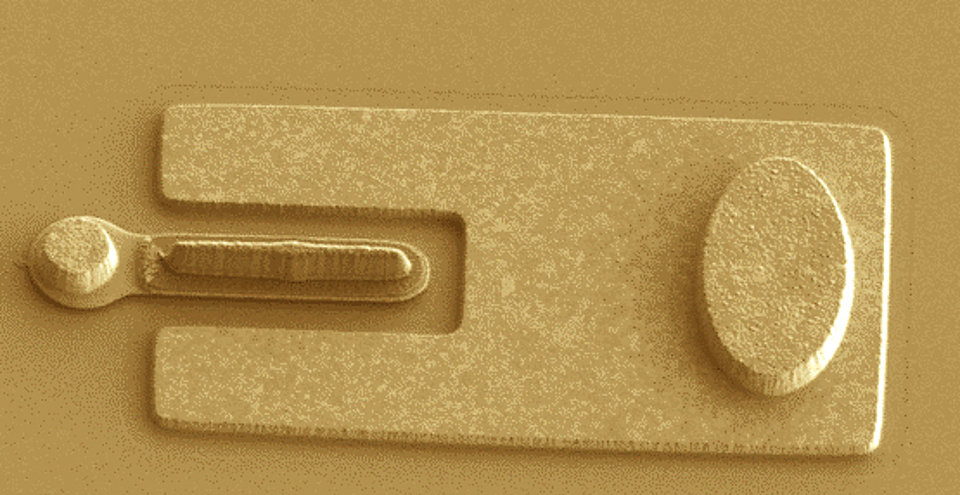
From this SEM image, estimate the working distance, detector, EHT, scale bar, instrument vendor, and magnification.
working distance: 7.9 mm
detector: SE(L)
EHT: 1 kV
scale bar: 5000 nm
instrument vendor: Hitachi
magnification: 6 K X